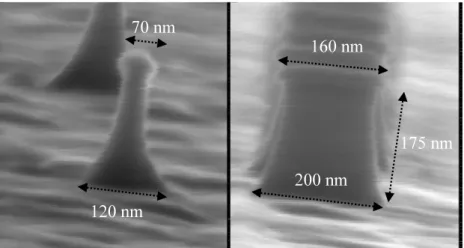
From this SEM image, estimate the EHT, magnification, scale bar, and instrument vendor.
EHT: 25 kV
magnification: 300 K X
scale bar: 100 nm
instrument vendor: JEOL